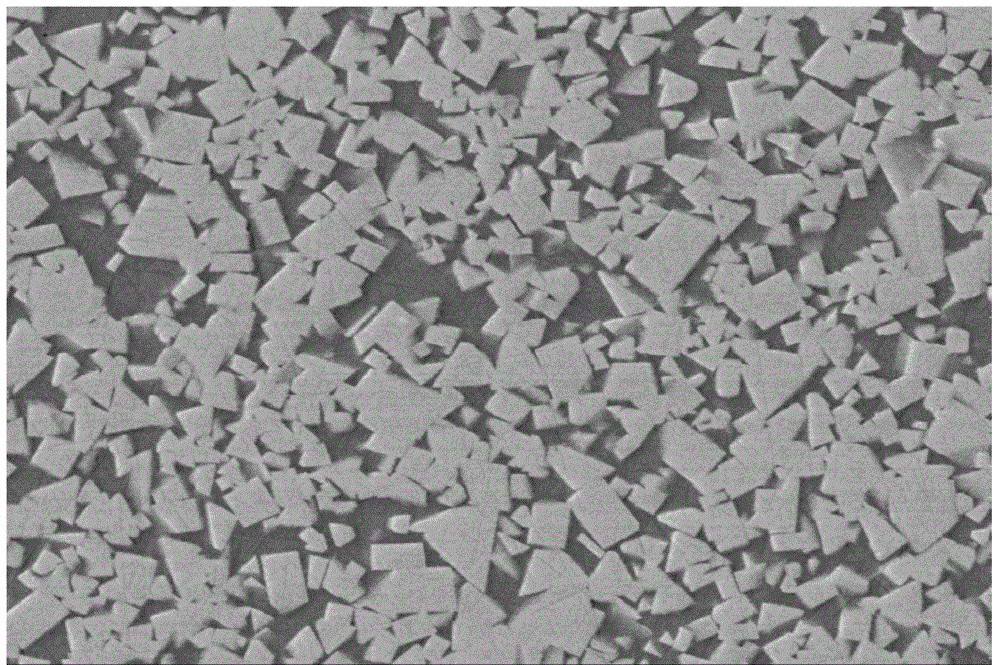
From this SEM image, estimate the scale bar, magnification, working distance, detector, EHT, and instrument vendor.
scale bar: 1000 nm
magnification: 3 K X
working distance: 14.4 mm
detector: LEI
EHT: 15 kV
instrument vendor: JEOL